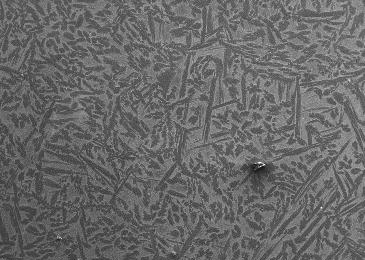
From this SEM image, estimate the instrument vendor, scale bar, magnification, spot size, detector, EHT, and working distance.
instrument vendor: JEOL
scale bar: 500000 nm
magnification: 0.037 K X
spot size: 60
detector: SEI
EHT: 20 kV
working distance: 10 mm